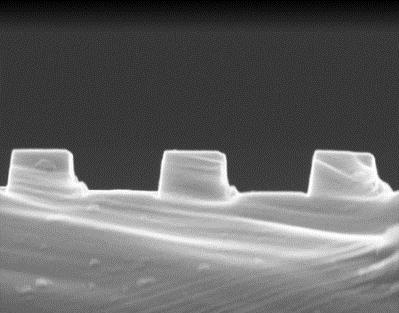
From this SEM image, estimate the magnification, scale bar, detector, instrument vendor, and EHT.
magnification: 35 K X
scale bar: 500 nm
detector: ETD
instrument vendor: FEI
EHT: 30 kV